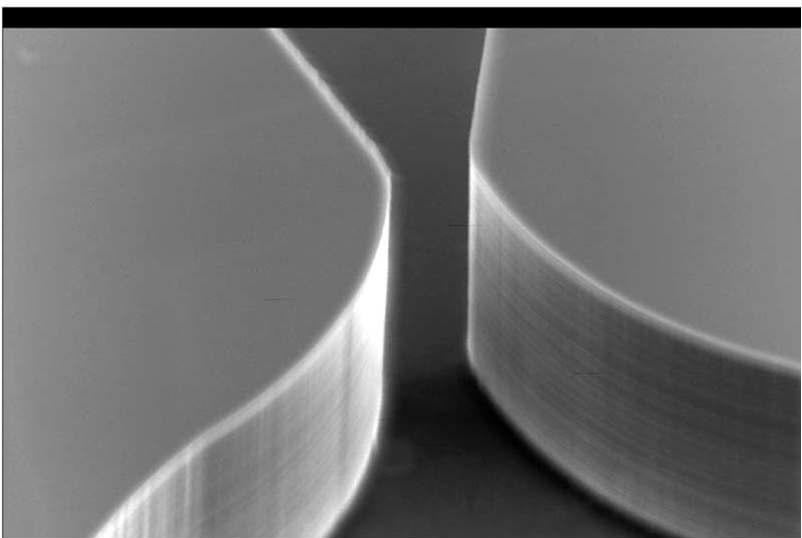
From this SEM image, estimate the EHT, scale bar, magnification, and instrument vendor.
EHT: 15 kV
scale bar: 10000 nm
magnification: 1.5 K X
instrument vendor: JEOL